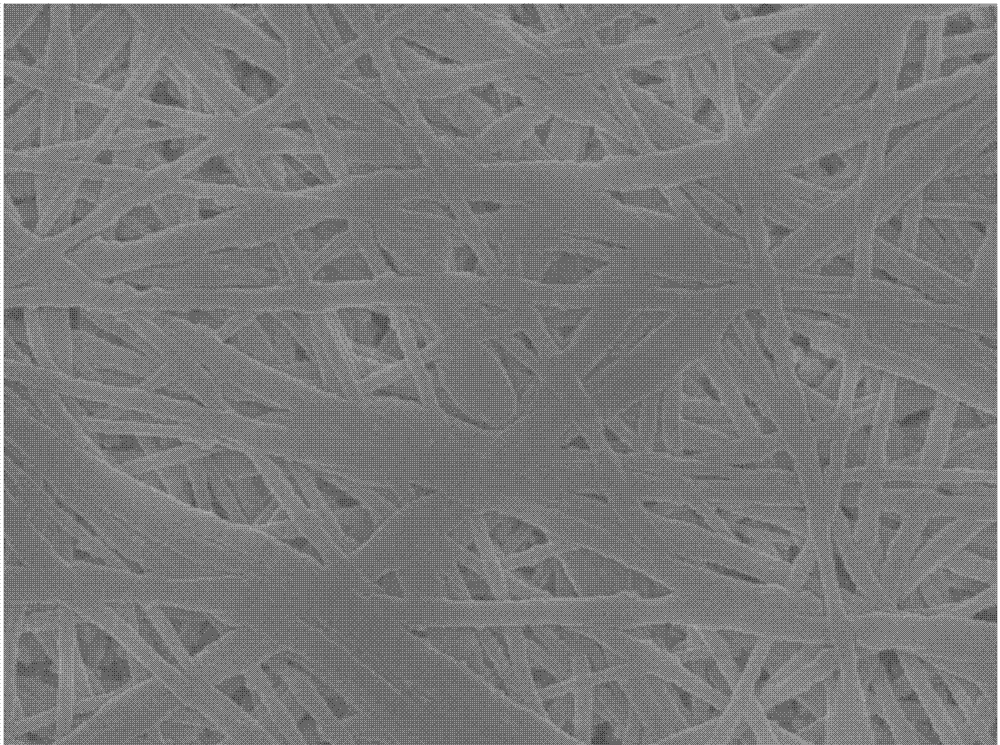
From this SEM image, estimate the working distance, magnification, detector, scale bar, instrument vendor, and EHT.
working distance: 7.8 mm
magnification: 5 K X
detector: SEM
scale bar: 1000 nm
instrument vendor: JEOL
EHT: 15 kV